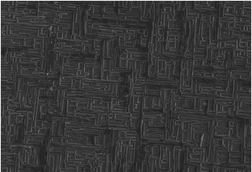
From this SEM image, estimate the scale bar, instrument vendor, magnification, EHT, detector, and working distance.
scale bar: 10000 nm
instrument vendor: Hitachi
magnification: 3.5 K X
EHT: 5 kV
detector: SE(UL)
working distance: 9.2 mm